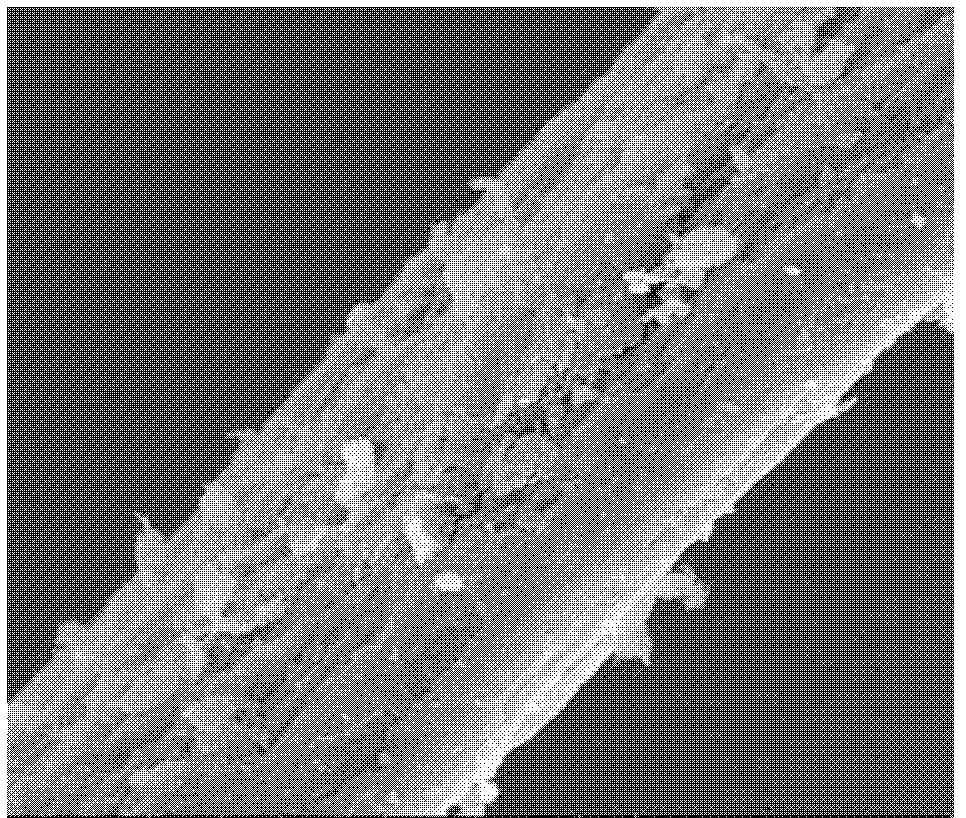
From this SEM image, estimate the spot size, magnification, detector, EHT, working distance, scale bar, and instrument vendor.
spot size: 3.5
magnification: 5 K X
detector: ETD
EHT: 12.5 kV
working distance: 10.9 mm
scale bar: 5000 nm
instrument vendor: FEI